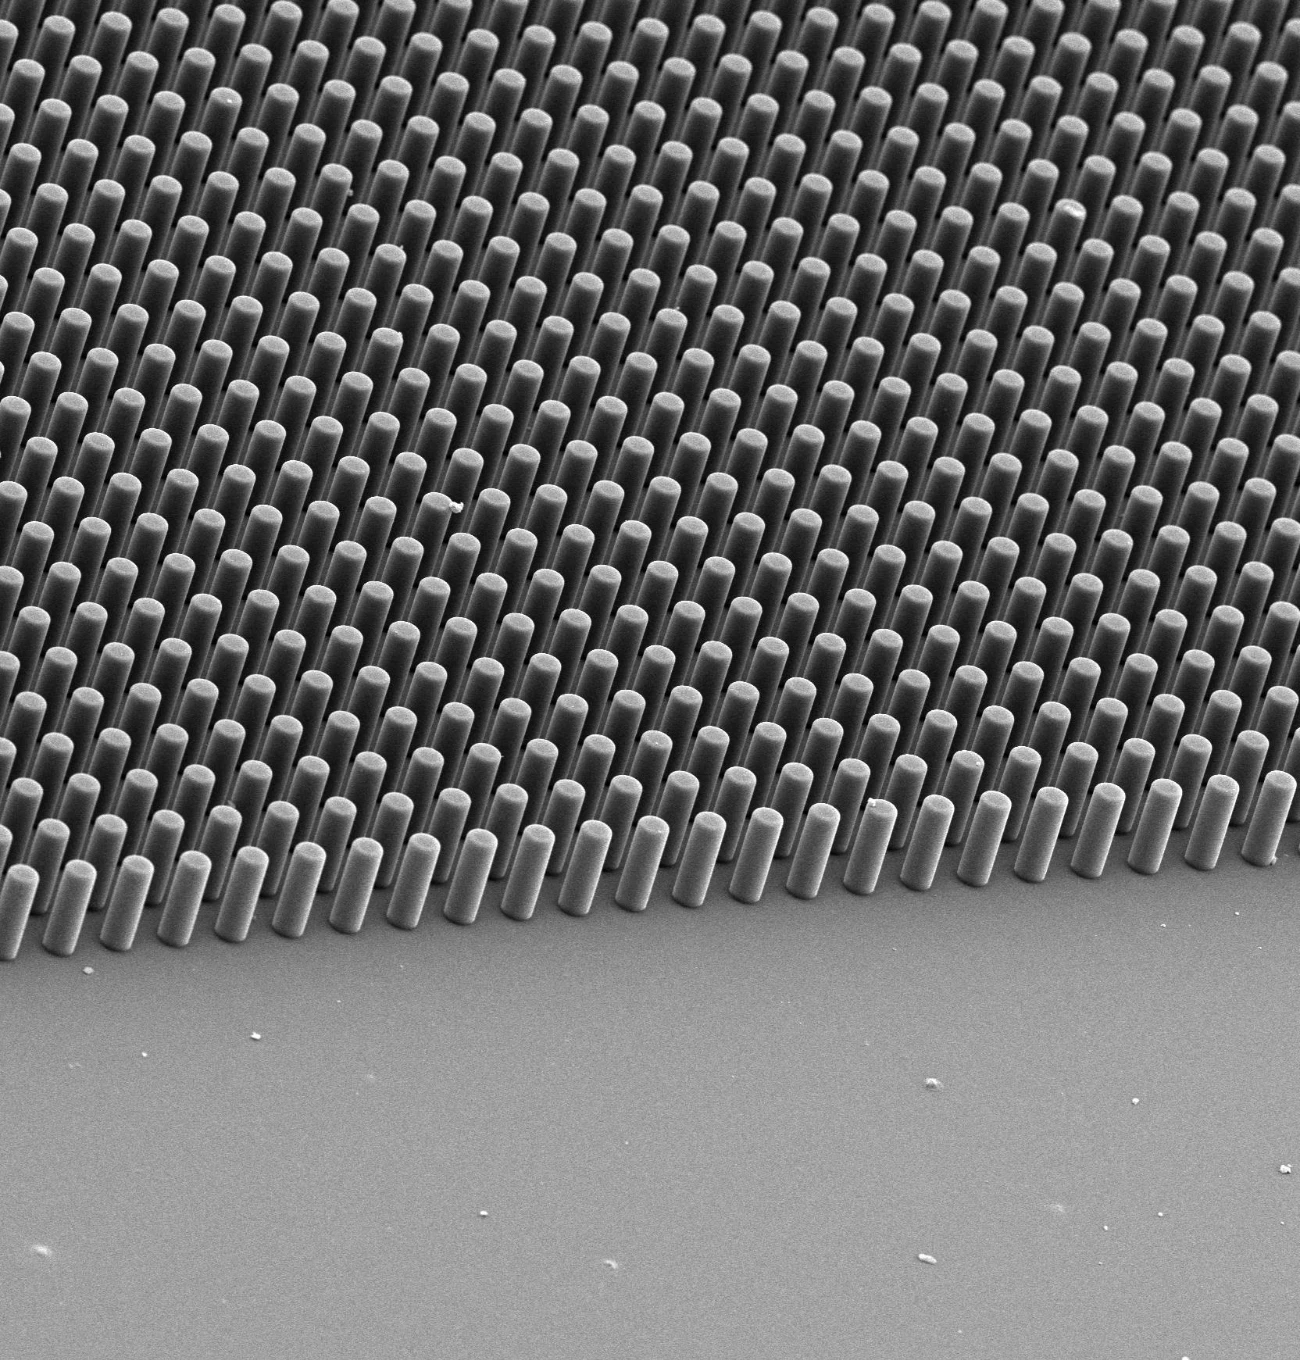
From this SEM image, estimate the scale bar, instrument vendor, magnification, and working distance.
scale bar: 100000 nm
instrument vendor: JEOL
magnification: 0.22 K X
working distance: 26 mm